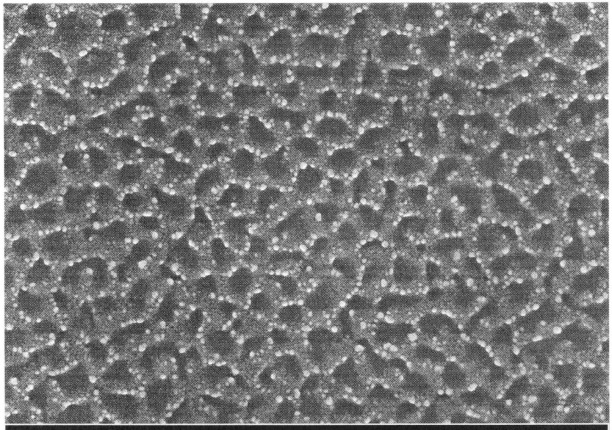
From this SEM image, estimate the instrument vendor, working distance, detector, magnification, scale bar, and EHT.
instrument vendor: Zeiss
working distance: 2.1 mm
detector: InLens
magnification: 25 K X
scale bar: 200 nm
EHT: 15 kV